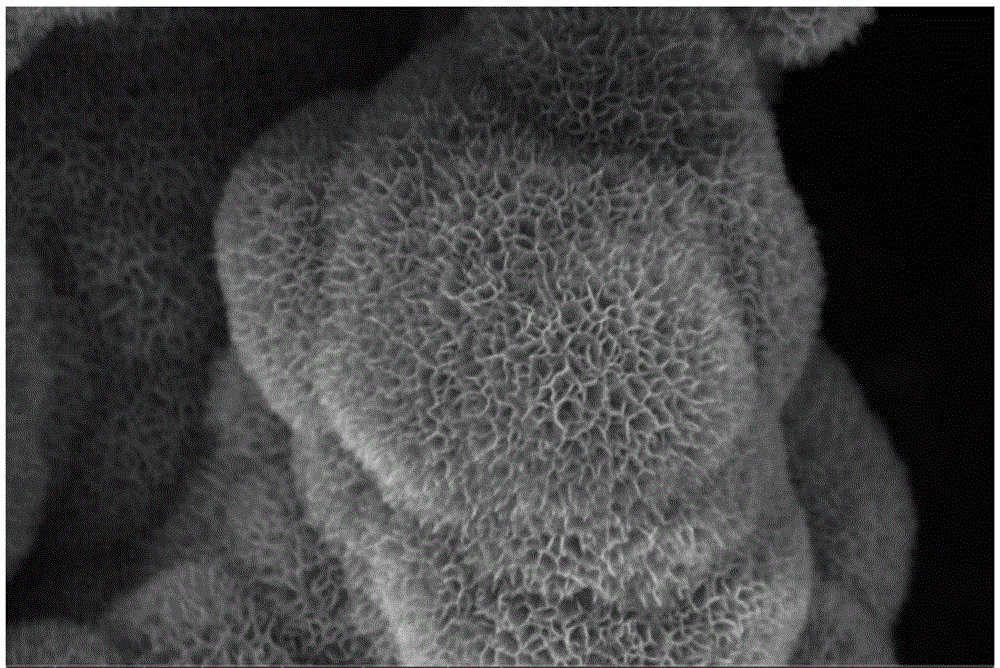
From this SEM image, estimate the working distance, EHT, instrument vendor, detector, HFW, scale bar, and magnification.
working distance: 5.1 mm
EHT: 5 kV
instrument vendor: FEI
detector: TLD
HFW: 4.14 µm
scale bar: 1000 nm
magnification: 50 K X